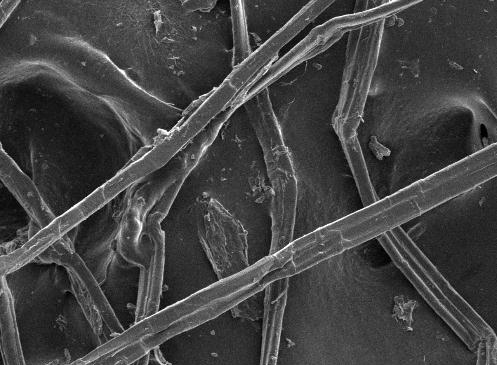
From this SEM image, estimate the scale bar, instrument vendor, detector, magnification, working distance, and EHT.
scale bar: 10000 nm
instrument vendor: JEOL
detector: SEI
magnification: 0.3 K X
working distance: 8 mm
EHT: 5 kV